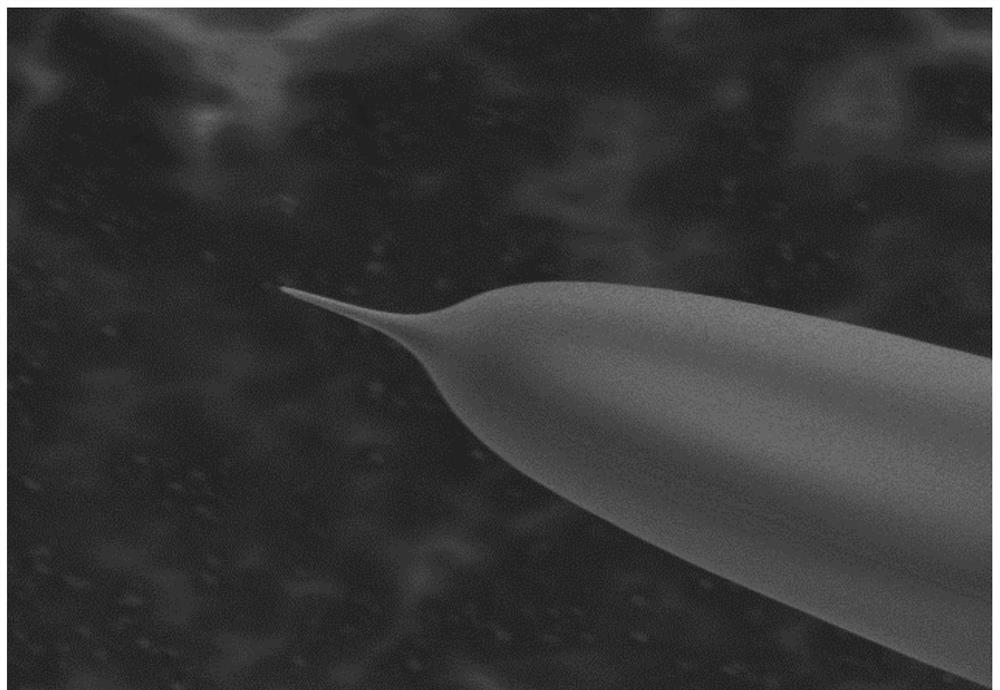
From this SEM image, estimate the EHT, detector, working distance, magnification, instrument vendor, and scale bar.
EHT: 3 kV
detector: SE(M)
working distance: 10 mm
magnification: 8 K X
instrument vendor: Hitachi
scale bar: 5000 nm